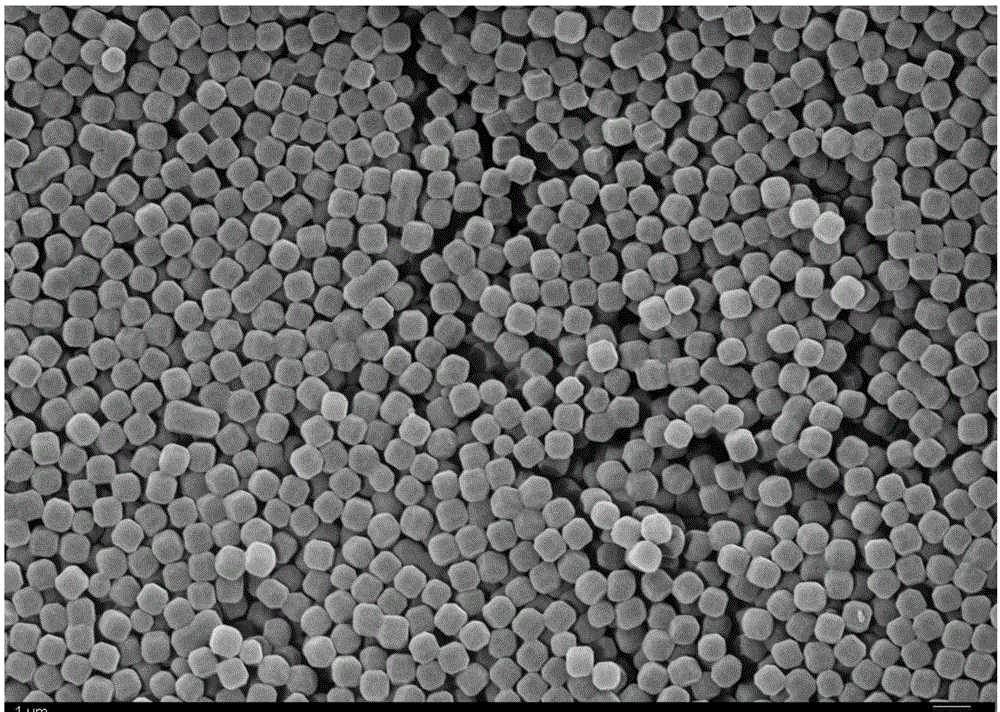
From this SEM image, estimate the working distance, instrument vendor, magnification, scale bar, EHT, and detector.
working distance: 10.9 mm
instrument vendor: Zeiss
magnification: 30 K X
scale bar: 1000 nm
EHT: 15 kV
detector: SE2